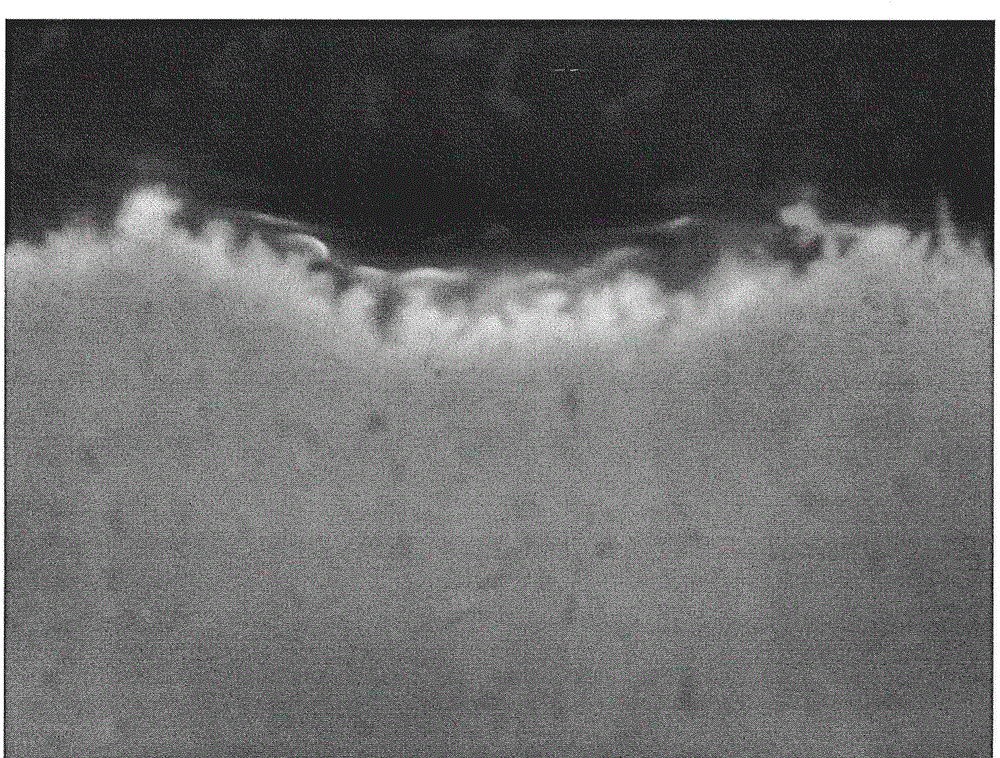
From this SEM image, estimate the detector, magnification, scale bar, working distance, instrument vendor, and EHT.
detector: LED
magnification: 50 K X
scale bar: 100 nm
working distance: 15 mm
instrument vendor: JEOL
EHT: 7 kV